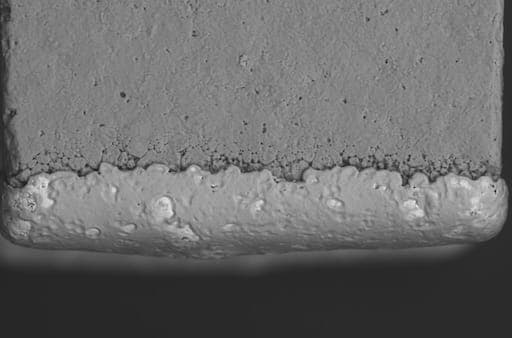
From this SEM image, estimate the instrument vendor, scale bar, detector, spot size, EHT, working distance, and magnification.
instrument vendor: JEOL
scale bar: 50000 nm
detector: BES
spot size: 60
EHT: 20 kV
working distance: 10 mm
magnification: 0.45 K X